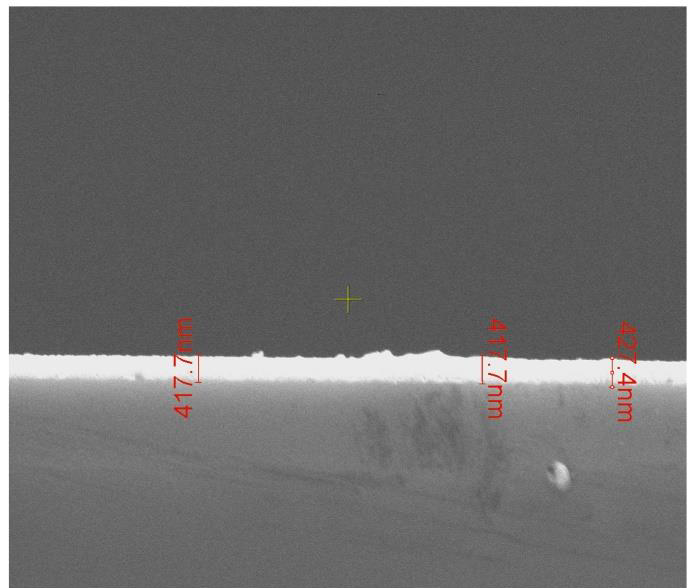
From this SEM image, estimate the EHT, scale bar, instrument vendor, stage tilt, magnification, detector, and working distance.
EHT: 10 kV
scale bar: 3000 nm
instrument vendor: FEI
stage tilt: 50°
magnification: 30 K X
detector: ETD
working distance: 7.2 mm